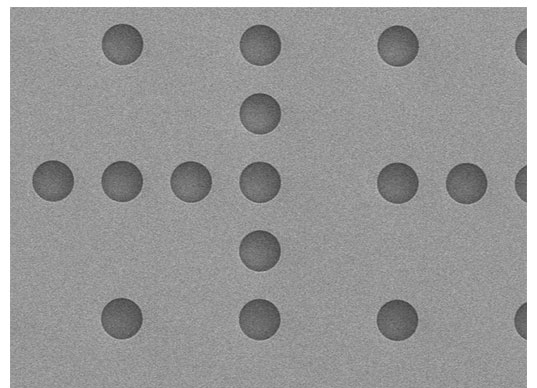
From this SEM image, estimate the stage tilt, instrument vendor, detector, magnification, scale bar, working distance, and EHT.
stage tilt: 0°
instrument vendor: JEOL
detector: LED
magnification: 0.65 K X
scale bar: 10000 nm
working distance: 10.2 mm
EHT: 5 kV